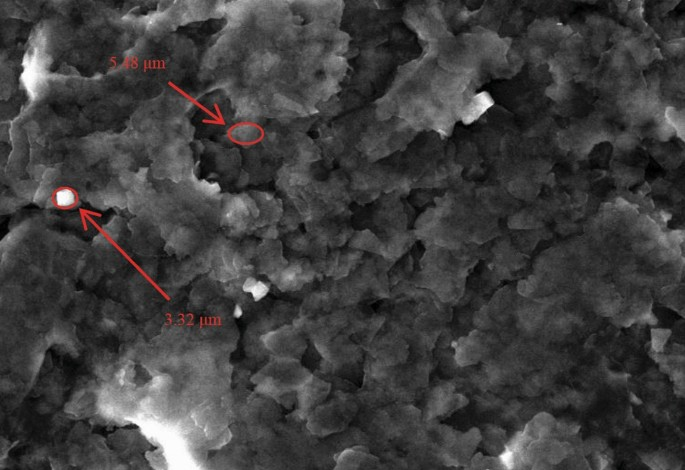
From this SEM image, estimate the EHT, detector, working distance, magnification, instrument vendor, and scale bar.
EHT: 20 kV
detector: SED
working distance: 10.4 mm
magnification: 1 K X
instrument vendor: JEOL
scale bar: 10000 nm